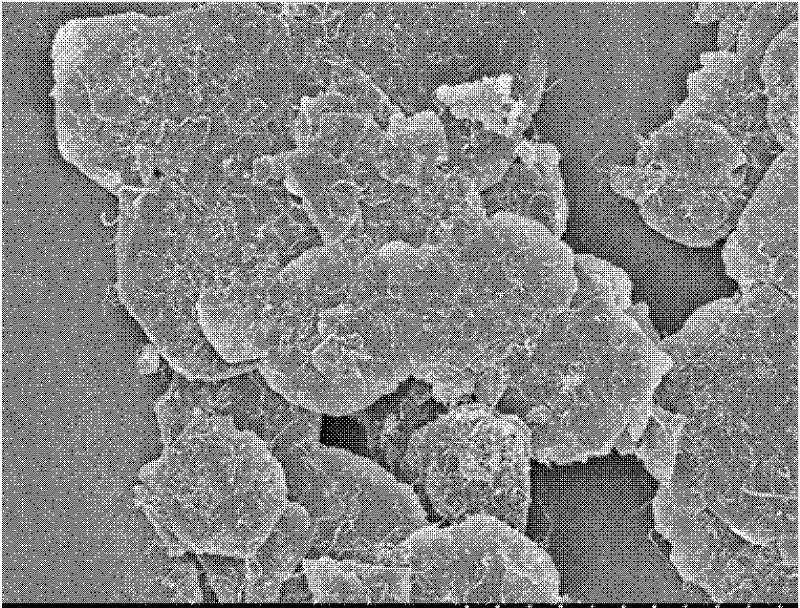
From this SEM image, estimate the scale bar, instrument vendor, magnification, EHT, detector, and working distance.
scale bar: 10000 nm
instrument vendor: Hitachi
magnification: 5 K X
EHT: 3 kV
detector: SE(M)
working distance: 8.2 mm